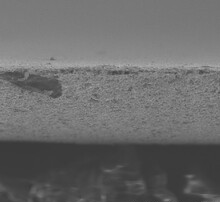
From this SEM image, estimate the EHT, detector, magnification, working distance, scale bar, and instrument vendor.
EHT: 10 kV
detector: SE(UL)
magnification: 1 K X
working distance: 9.9 mm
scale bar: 50000 nm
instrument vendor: Hitachi